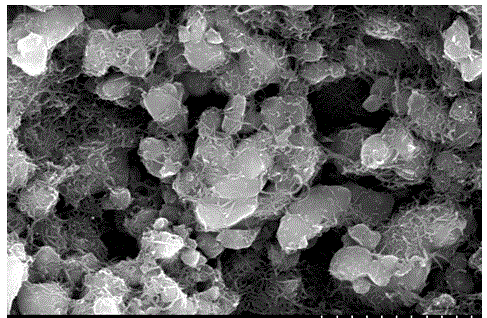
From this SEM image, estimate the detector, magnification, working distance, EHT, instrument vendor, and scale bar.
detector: SE(M)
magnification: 20 K X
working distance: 12.7 mm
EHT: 15 kV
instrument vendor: Hitachi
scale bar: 2000 nm